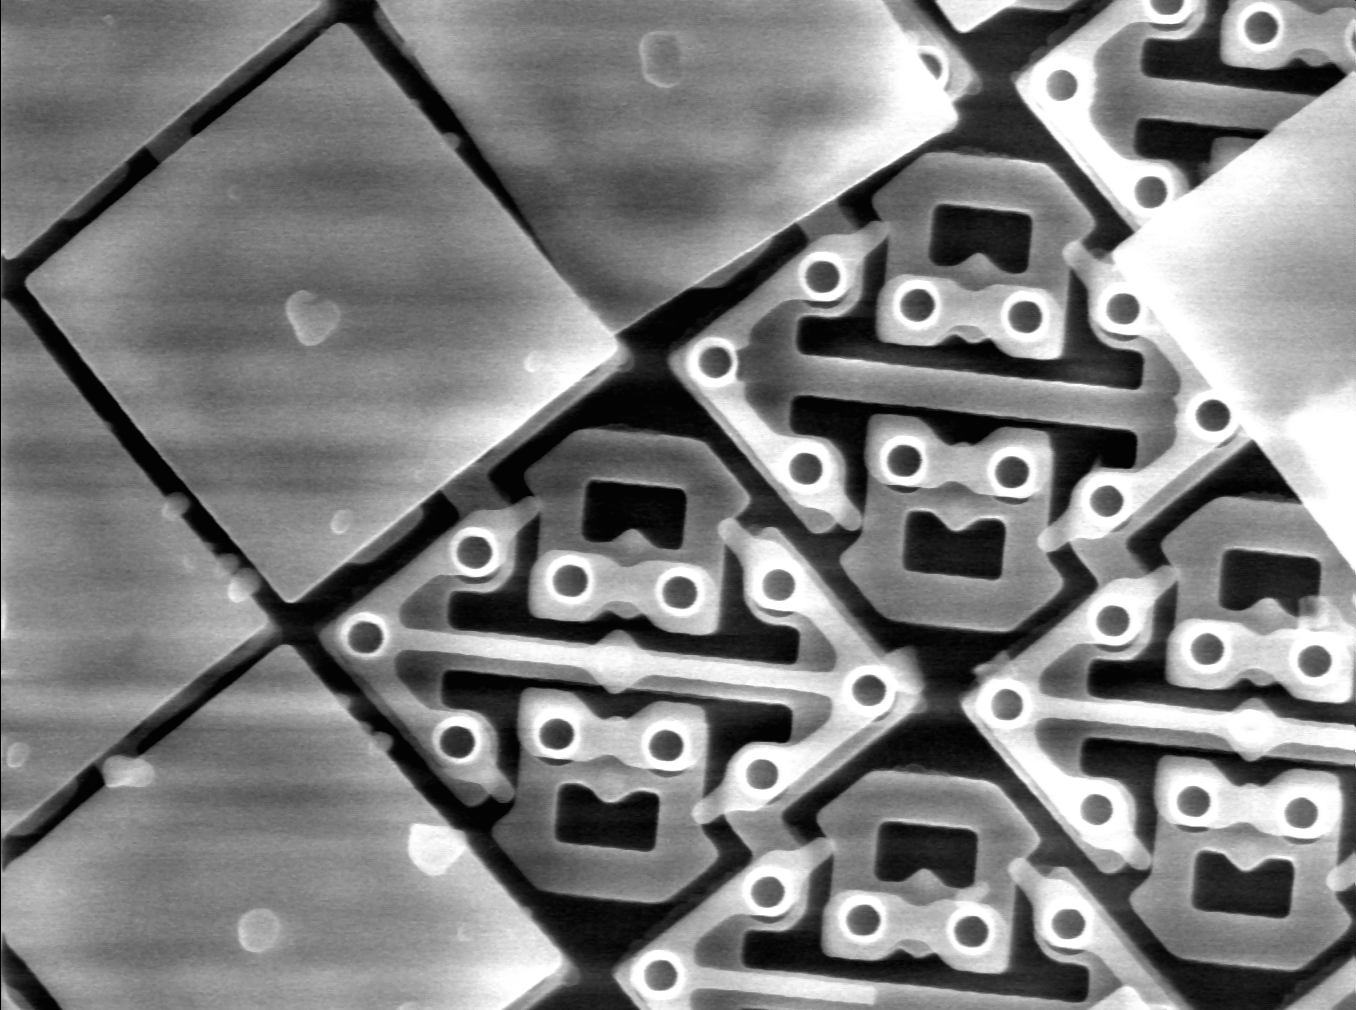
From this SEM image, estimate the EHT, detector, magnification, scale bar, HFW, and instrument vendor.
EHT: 15 kV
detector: SE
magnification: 3 K X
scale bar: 3330 nm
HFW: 40 µm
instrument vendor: other